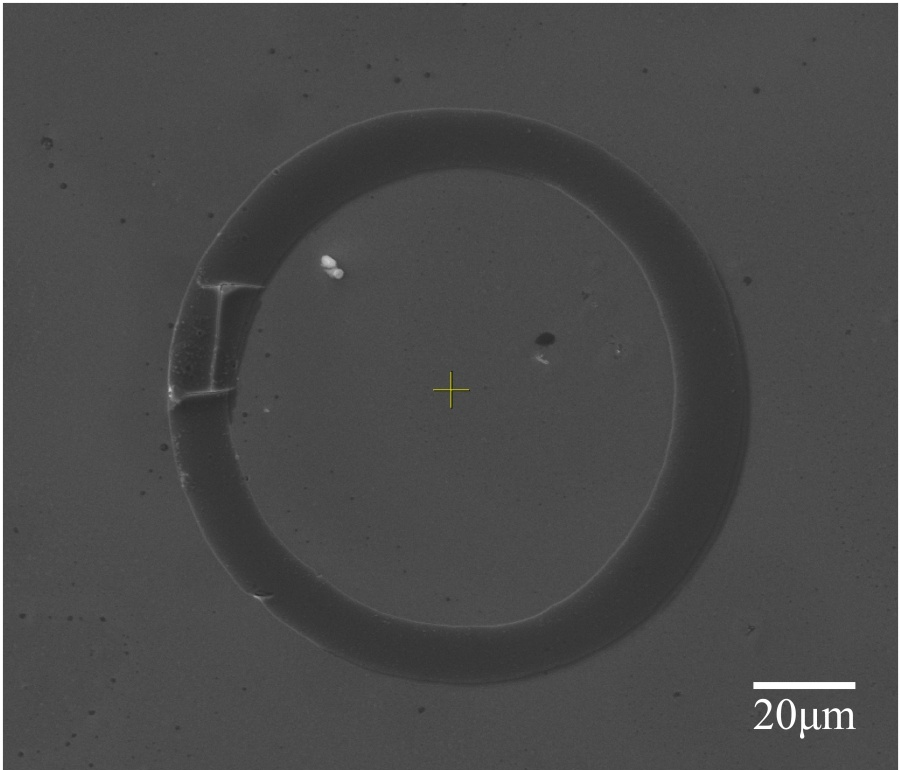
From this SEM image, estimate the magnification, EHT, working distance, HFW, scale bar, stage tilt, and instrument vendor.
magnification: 1.535 K X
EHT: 20 kV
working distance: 15 mm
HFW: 176 µm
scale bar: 50000 nm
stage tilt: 0°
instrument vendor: FEI